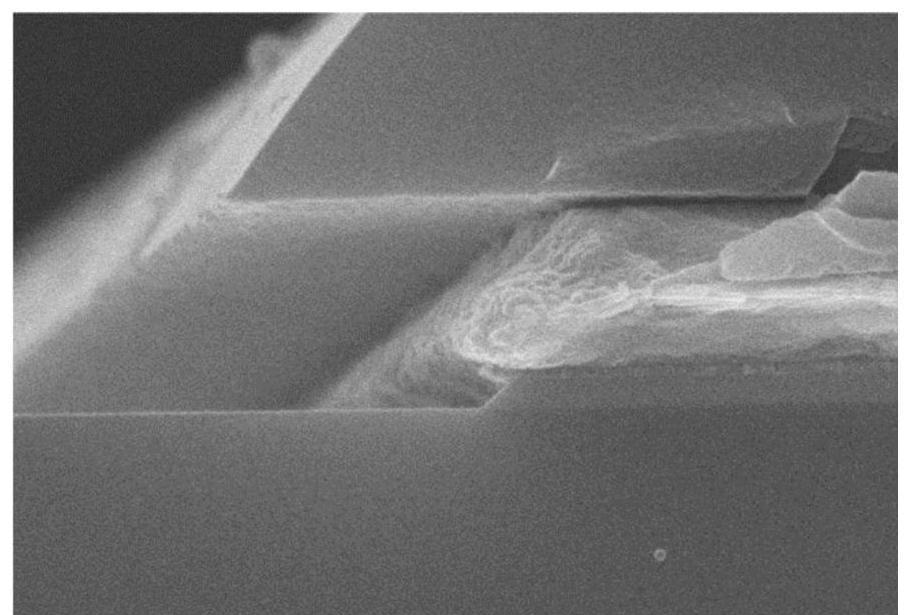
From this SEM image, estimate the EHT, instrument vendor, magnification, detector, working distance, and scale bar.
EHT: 10 kV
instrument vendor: Hitachi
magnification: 50 K X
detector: SE(U)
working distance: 11.9 mm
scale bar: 1000 nm